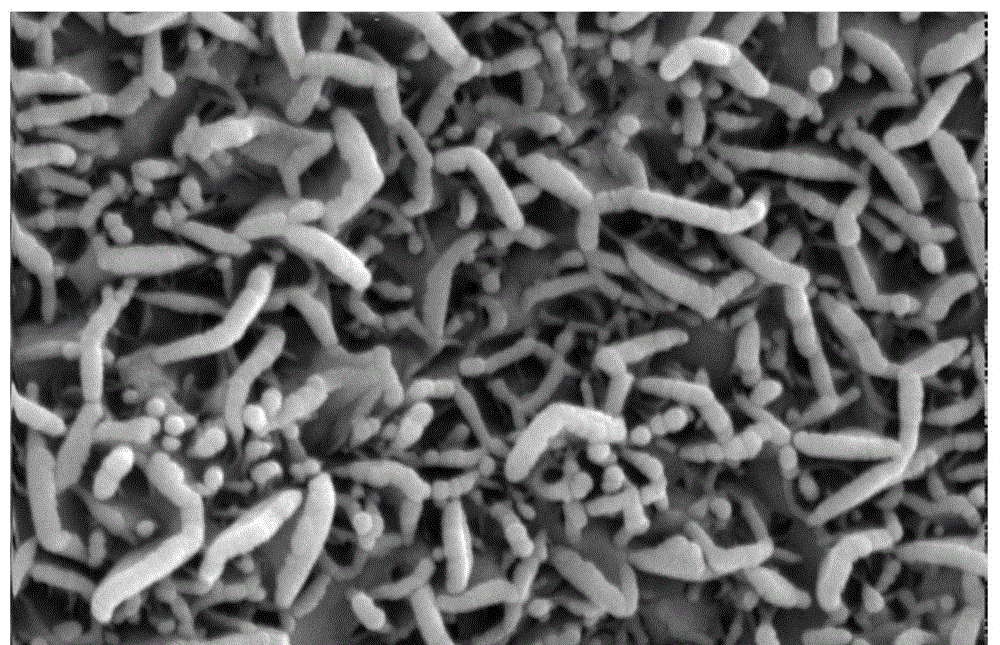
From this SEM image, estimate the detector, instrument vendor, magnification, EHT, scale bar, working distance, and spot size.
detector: SE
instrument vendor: FEI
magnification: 50 K X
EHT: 20 kV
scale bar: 1000 nm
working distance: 7 mm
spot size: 3